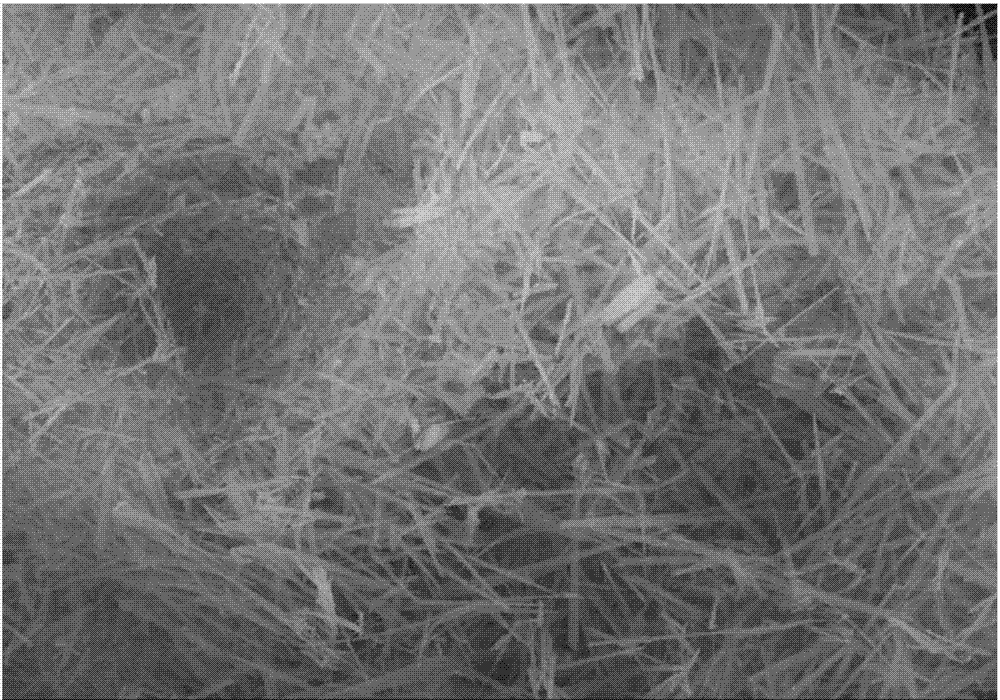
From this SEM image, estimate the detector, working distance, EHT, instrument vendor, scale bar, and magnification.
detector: SE(M)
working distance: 14.7 mm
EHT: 15 kV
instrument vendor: Hitachi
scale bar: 10000 nm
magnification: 5 K X